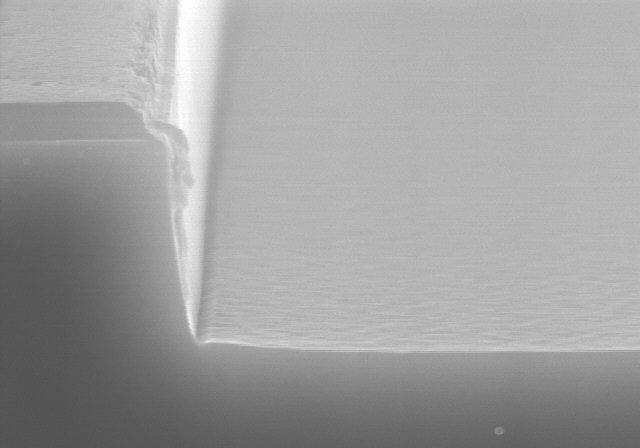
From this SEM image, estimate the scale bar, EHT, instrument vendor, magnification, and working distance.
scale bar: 1000 nm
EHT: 15 kV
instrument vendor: Hitachi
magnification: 30 K X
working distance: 11.7 mm